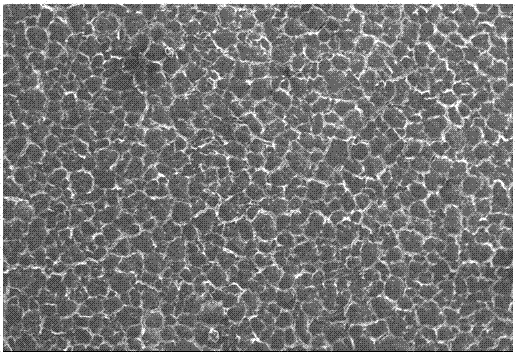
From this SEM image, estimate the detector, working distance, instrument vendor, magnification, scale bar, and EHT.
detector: SEI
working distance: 10 mm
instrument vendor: JEOL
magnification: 0.1 K X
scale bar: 100000 nm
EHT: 10 kV